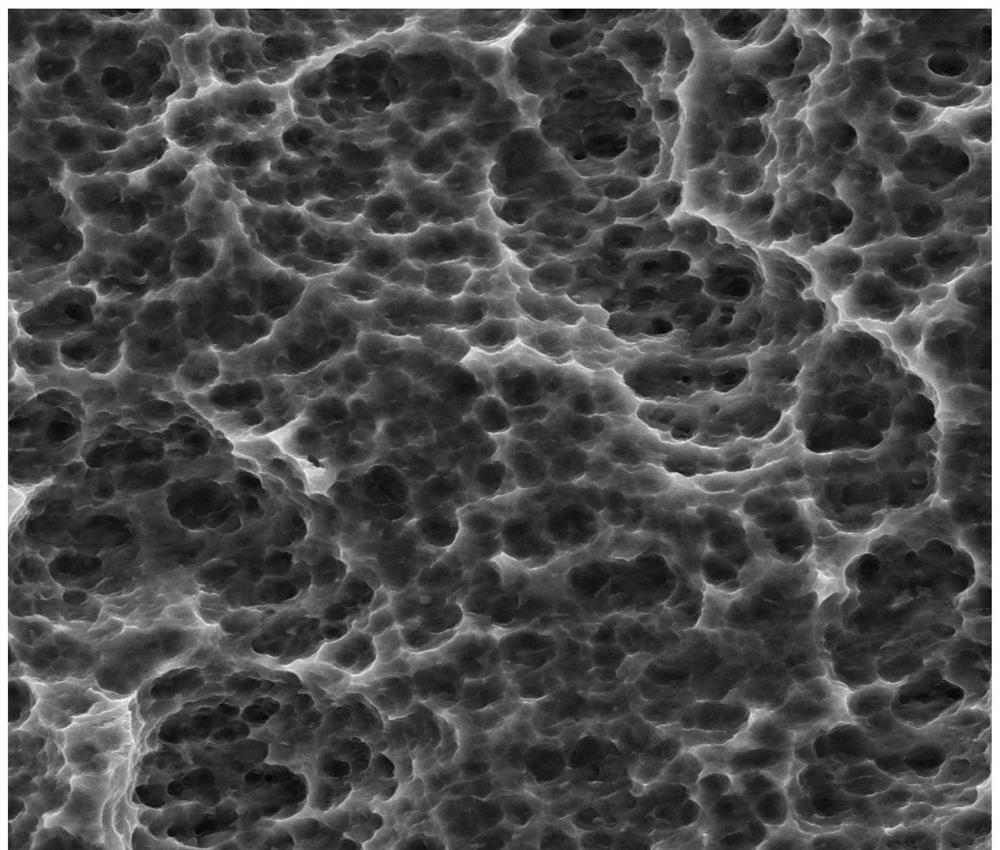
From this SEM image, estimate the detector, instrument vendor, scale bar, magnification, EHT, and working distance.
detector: ETD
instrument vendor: FEI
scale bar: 20000 nm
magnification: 6 K X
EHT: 20 kV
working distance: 6.3 mm